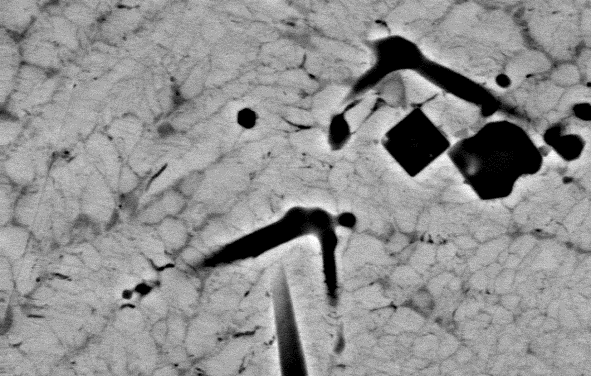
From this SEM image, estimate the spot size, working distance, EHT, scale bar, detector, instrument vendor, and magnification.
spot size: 4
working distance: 9 mm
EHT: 20 kV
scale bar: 5000 nm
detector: BSE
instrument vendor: FEI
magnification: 5 K X